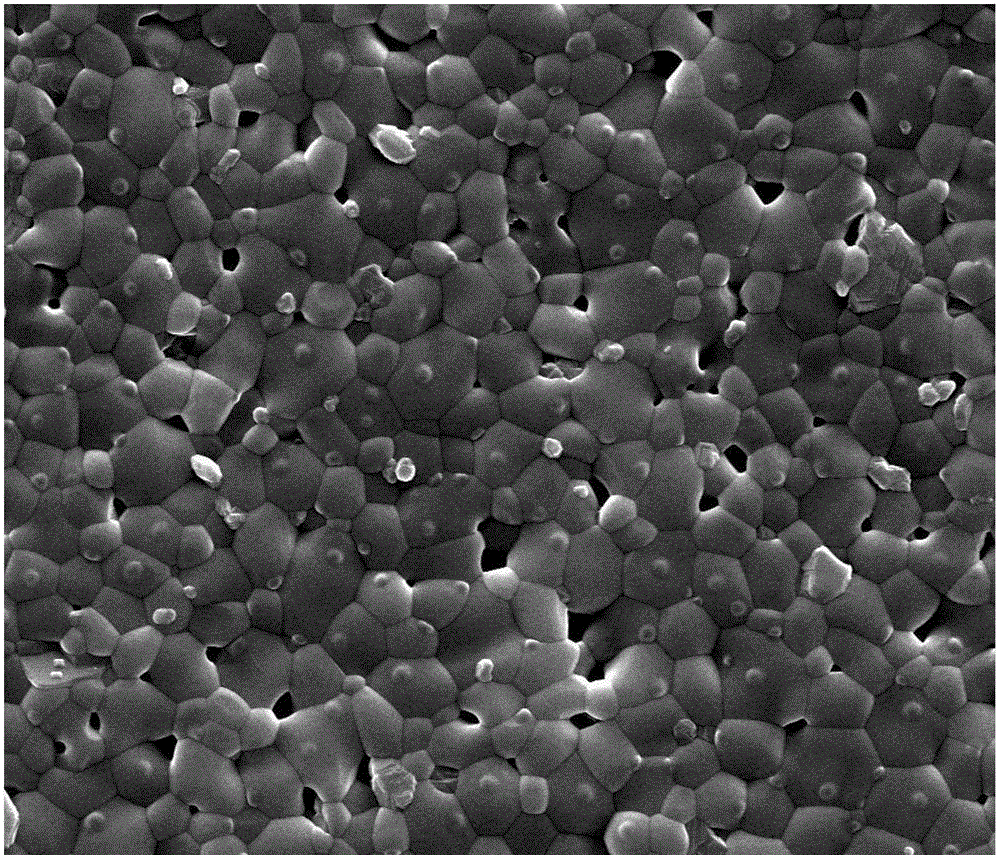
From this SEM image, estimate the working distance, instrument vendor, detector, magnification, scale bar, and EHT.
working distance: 11.2 mm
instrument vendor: FEI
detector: SE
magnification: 20 K X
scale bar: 5000 nm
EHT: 20 kV